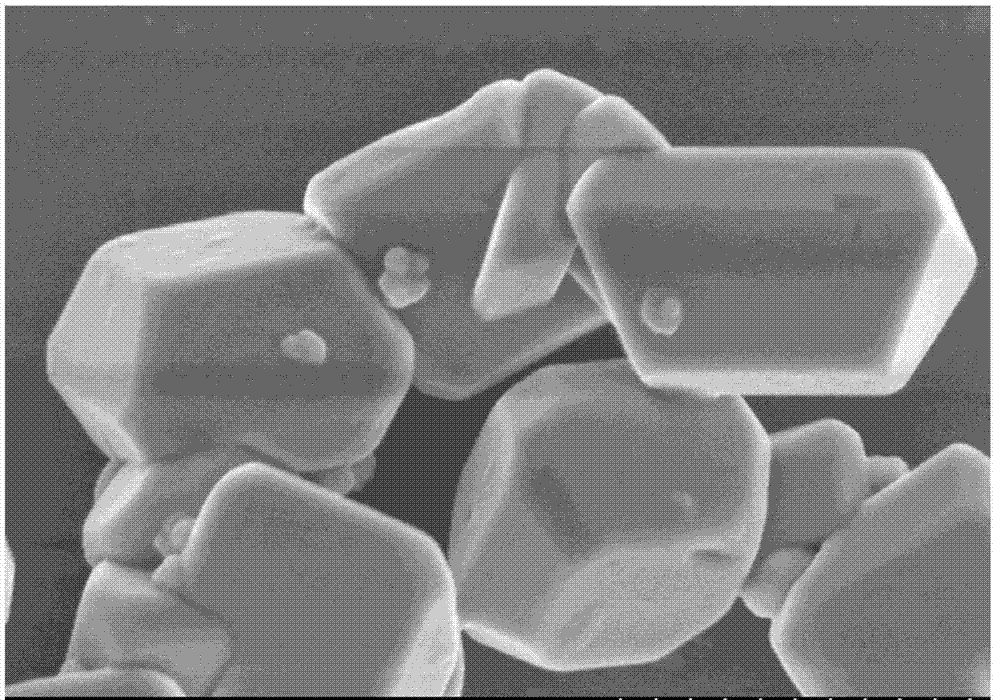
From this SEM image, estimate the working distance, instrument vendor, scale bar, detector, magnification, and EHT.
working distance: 4.5 mm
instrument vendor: Hitachi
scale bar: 1000 nm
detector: SE(M)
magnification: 45 K X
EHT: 3 kV